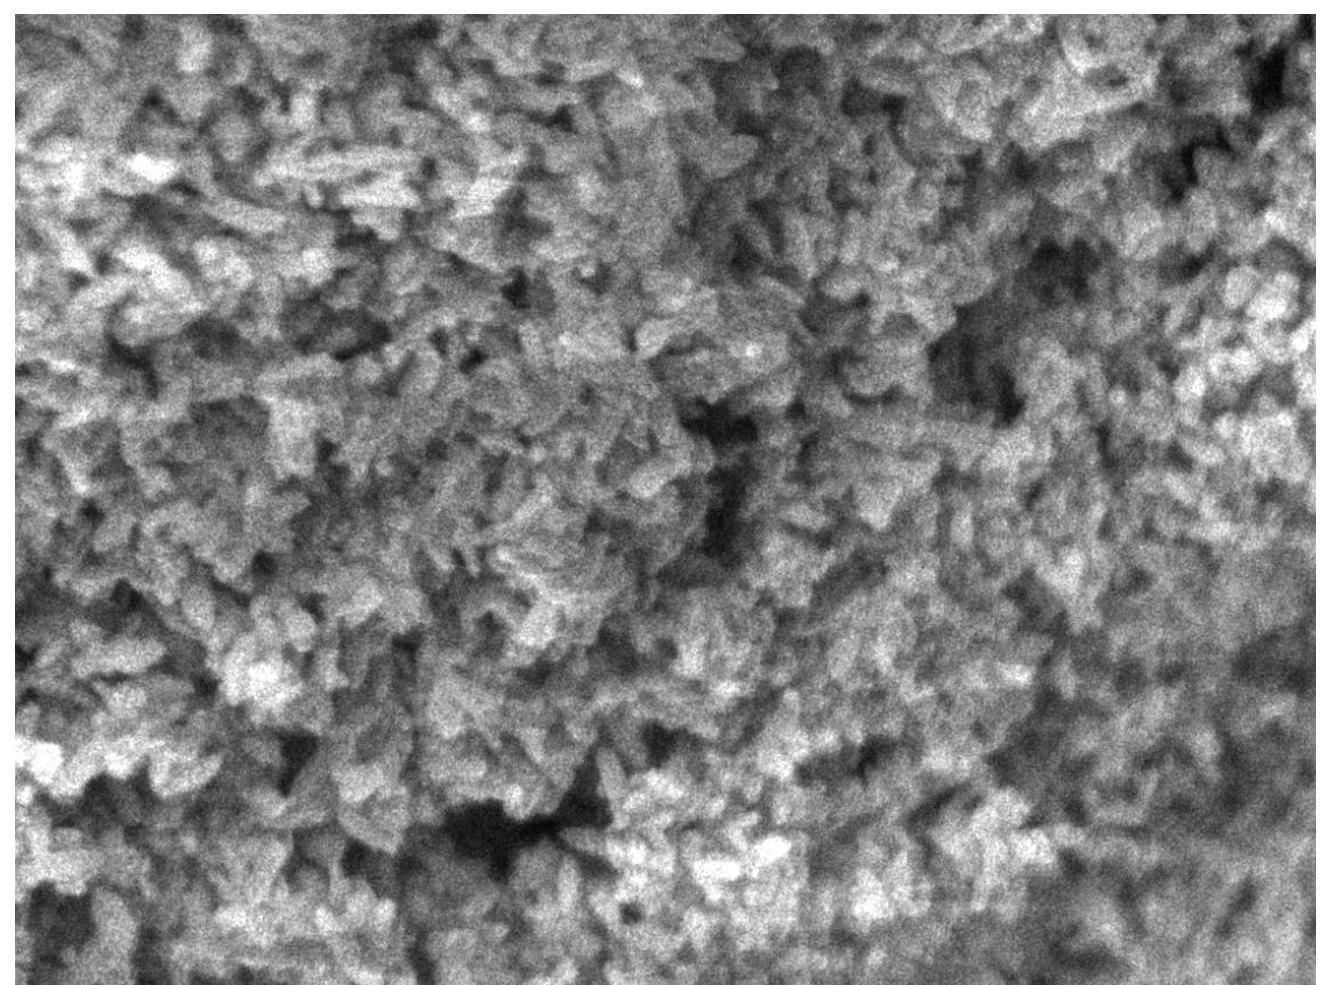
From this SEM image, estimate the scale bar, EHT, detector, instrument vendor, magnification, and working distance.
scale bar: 100 nm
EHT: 5 kV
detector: UED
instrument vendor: JEOL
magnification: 70 K X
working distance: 3.1 mm